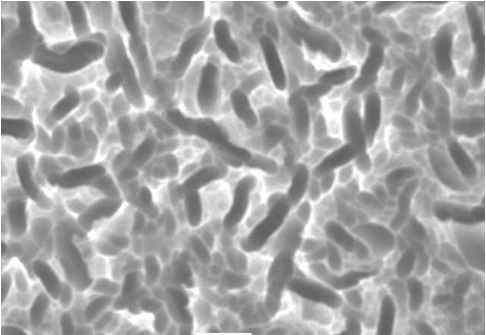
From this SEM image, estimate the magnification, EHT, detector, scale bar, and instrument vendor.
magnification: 3 K X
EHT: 20 kV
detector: SEI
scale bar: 5000 nm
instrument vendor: JEOL